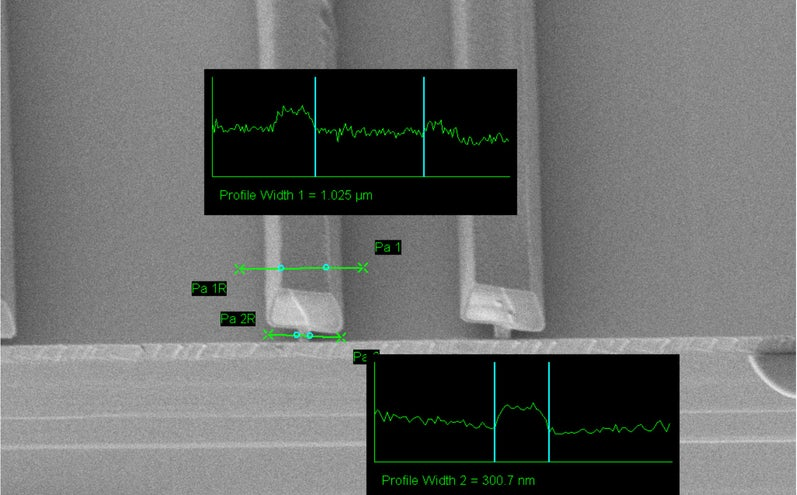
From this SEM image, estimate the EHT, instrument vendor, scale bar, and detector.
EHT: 2 kV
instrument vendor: Zeiss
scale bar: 1000 nm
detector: SE2